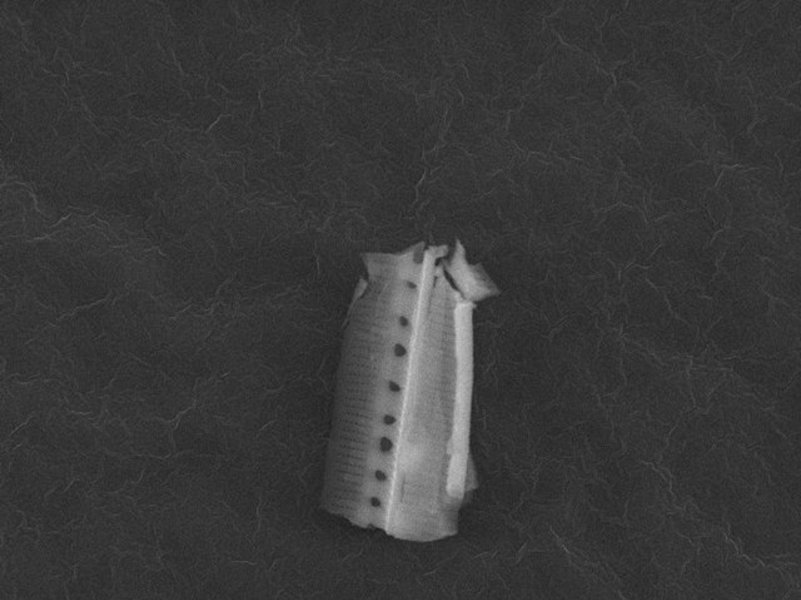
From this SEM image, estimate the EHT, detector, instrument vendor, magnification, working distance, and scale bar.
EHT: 15 kV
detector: SEI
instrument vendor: JEOL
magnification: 3.5 K X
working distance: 23 mm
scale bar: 1000 nm